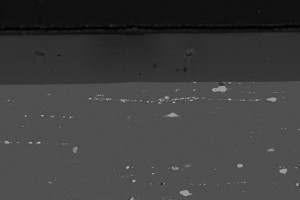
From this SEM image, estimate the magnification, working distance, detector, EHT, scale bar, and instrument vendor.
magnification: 0.5 K X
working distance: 23 mm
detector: BSD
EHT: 20 kV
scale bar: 10000 nm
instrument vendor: Zeiss